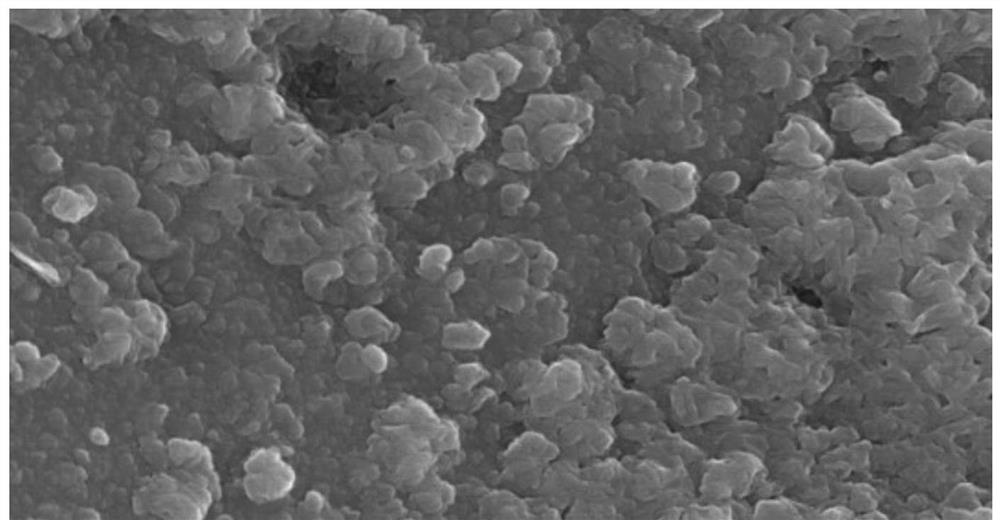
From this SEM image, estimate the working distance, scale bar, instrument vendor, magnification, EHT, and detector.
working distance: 8.3 mm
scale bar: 1000 nm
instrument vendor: Hitachi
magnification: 35 K X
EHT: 20 kV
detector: SE(M)